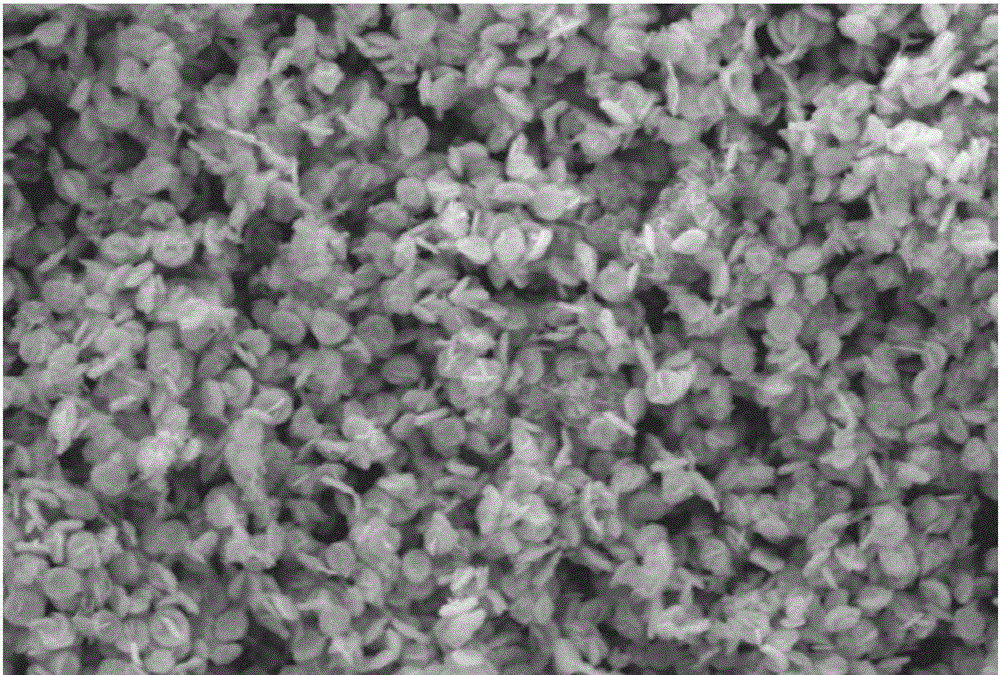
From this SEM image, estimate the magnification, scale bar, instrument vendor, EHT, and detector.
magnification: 5 K X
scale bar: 5000 nm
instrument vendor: JEOL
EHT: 15 kV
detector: SEI